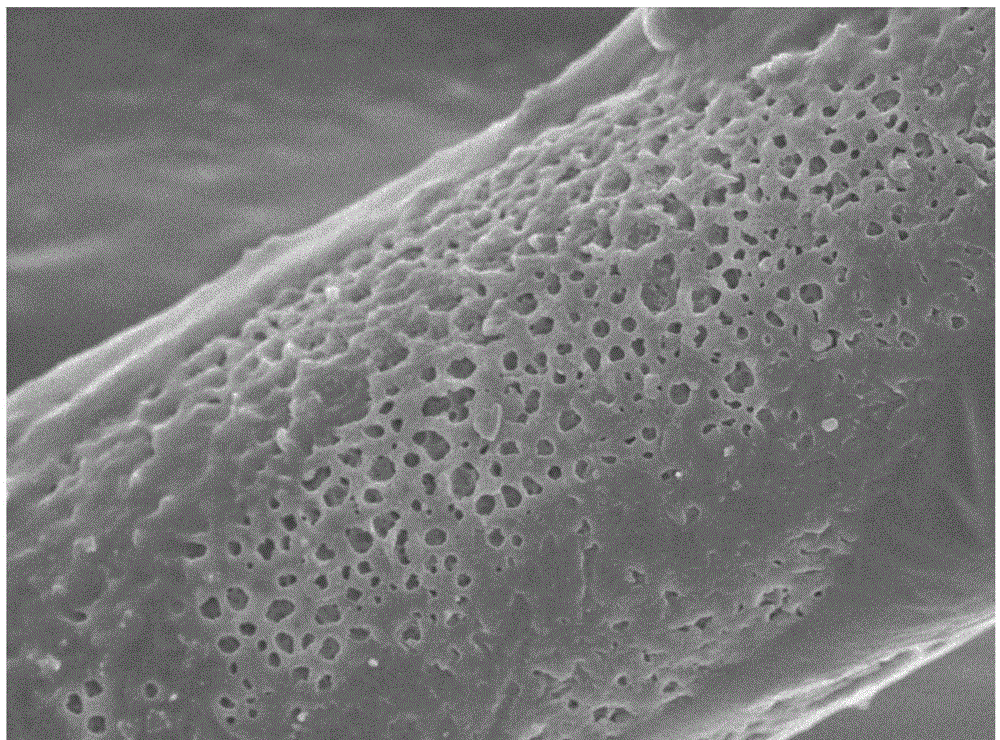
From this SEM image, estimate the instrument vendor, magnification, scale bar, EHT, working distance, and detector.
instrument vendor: JEOL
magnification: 8 K X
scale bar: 1000 nm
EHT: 5 kV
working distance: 8.2 mm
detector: SEI